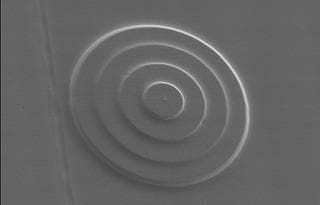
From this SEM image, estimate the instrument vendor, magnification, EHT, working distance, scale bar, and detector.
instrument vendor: JEOL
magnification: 1.5 K X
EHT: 5 kV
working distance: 15 mm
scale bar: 10000 nm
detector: SEMIMG